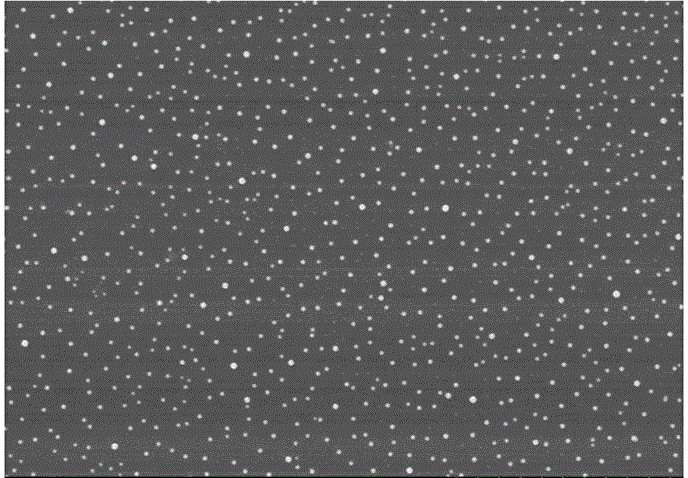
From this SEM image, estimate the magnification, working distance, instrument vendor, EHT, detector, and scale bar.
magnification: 20 K X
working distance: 8.3 mm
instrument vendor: Hitachi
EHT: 3 kV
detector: SE(U,LA0)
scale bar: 2000 nm